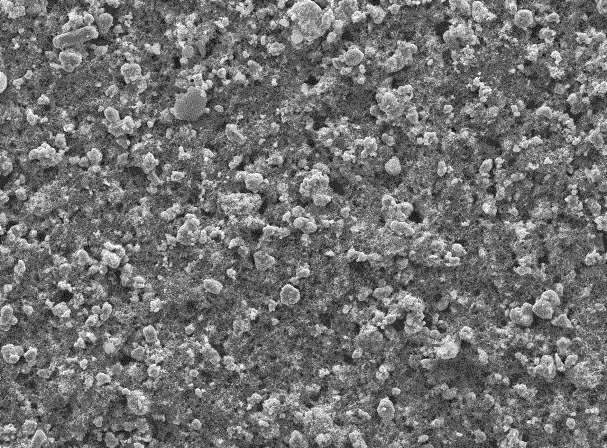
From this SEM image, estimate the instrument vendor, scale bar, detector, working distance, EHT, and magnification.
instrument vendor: JEOL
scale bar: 10000 nm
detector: SEI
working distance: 12.3 mm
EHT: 20 kV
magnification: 0.5 K X